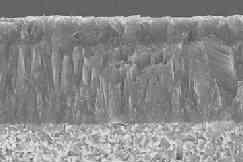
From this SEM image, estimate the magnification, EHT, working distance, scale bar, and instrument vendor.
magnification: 3 K X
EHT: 25 kV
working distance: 15 mm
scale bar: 10000 nm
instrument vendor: JEOL